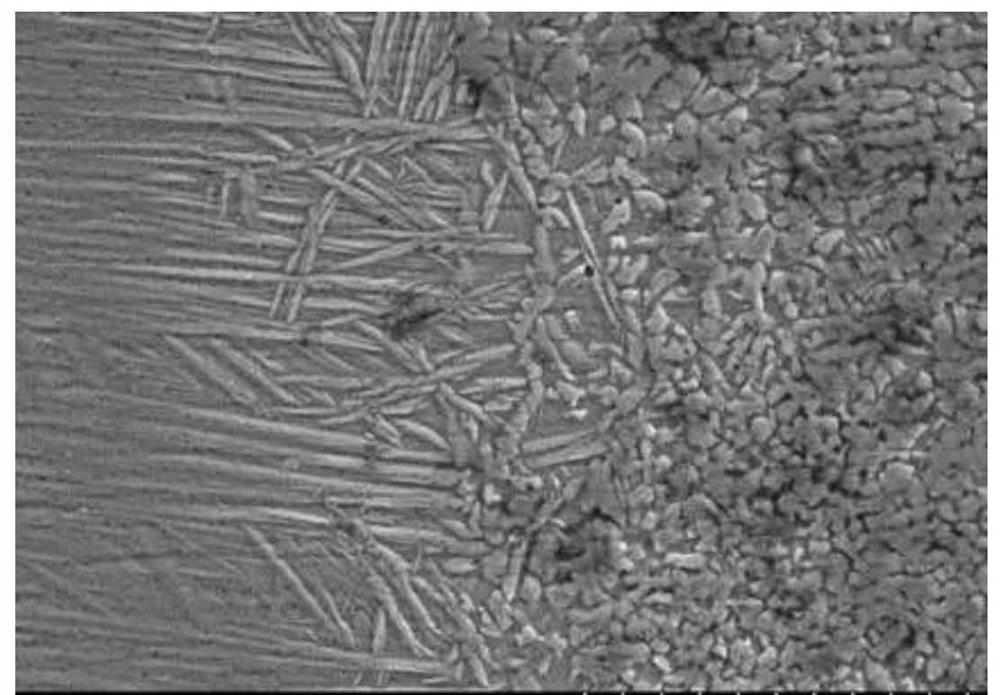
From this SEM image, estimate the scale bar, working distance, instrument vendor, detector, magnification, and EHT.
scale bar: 50000 nm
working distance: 11 mm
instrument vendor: Hitachi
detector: SE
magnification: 1 K X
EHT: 20 kV